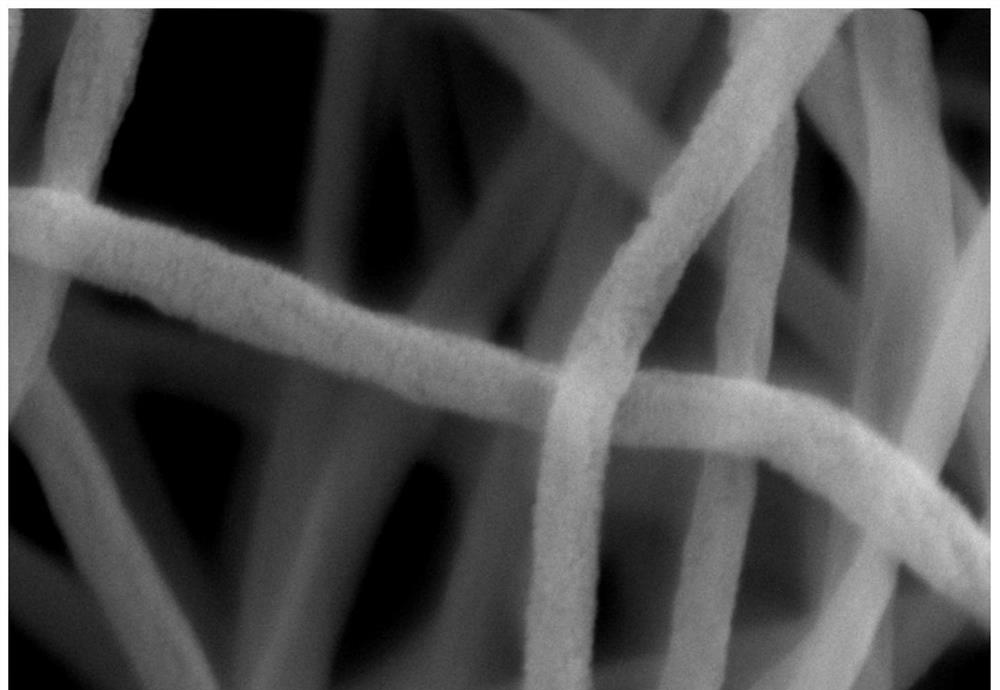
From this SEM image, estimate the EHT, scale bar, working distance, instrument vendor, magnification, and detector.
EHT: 10 kV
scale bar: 100 nm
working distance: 8.6 mm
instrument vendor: Zeiss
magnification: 100 K X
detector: SE2